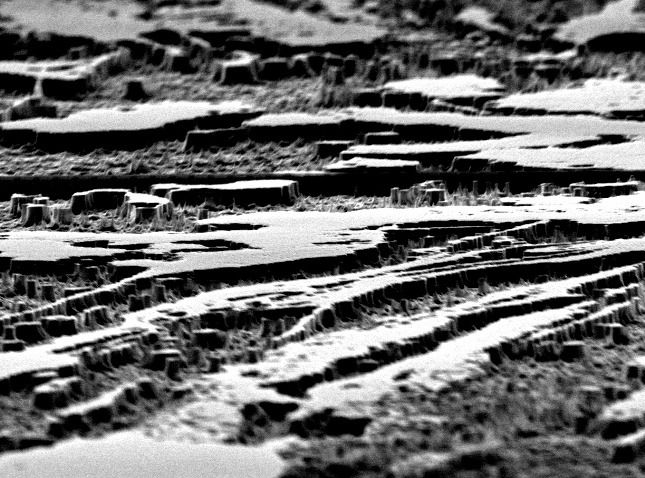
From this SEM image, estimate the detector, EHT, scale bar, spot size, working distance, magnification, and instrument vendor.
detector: SE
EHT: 30 kV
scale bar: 2000 nm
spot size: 3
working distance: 18.7 mm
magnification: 7.592 K X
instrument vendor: FEI